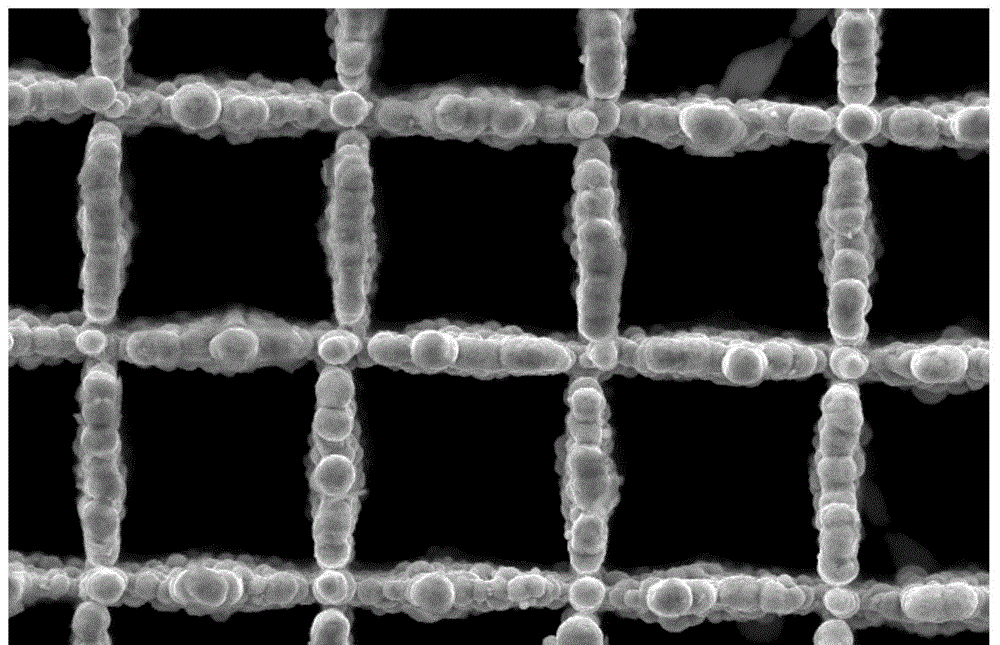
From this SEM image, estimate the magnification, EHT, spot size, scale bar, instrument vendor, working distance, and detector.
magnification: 5 K X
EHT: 10 kV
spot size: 3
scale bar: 5000 nm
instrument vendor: FEI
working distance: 6 mm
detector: SE